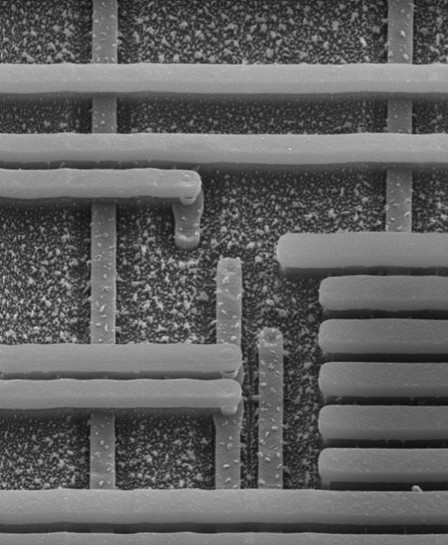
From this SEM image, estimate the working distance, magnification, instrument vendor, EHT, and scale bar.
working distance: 15 mm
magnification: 5 K X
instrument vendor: Zeiss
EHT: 20 kV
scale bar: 4000 nm